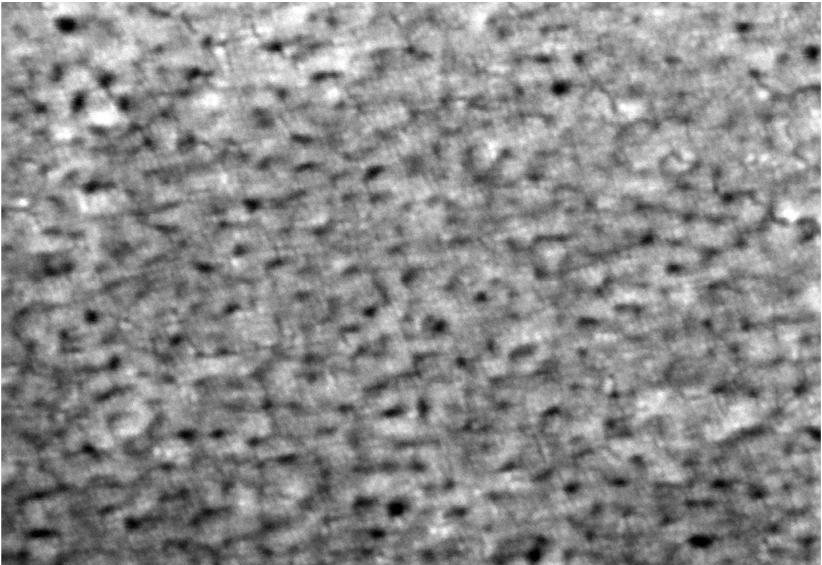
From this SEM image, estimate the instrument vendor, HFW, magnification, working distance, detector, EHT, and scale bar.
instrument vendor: Zeiss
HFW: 0.6027 µm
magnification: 500 K X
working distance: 4 mm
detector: InLens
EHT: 5 kV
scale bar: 20 nm